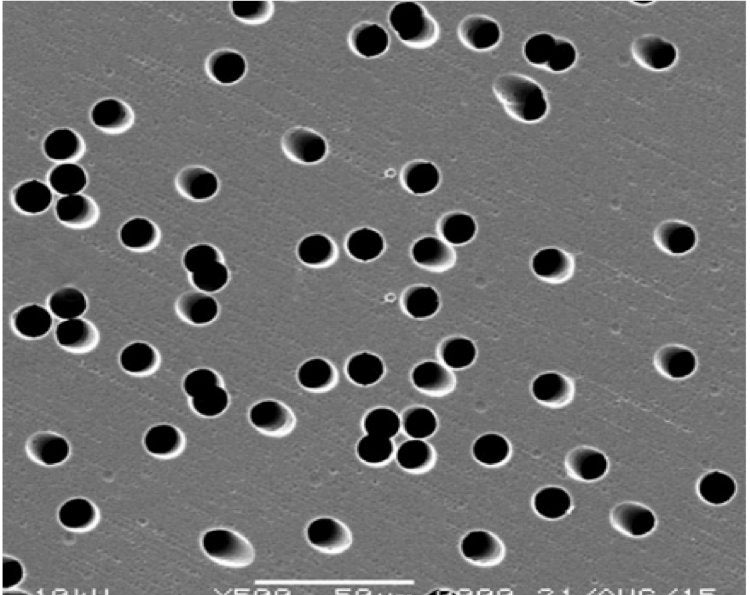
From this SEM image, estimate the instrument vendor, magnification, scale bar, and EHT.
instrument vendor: JEOL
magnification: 0.5 K X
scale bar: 50000 nm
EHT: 10 kV